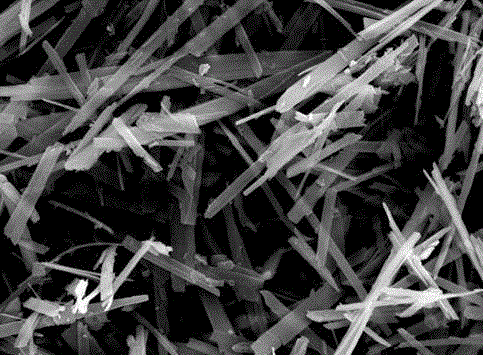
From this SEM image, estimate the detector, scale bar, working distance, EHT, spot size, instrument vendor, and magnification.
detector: SE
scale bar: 2000 nm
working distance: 15.2 mm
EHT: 10 kV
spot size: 3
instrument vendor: FEI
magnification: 10 K X